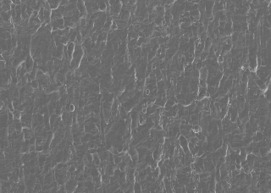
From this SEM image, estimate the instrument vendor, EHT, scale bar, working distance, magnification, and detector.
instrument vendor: JEOL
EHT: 10 kV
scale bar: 50000 nm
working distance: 12.1 mm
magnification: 0.5 K X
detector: SED